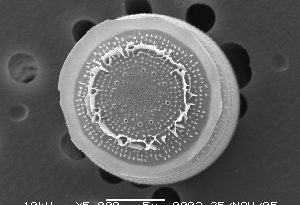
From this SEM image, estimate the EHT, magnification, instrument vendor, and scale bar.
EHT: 10 kV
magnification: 5 K X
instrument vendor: JEOL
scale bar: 5000 nm